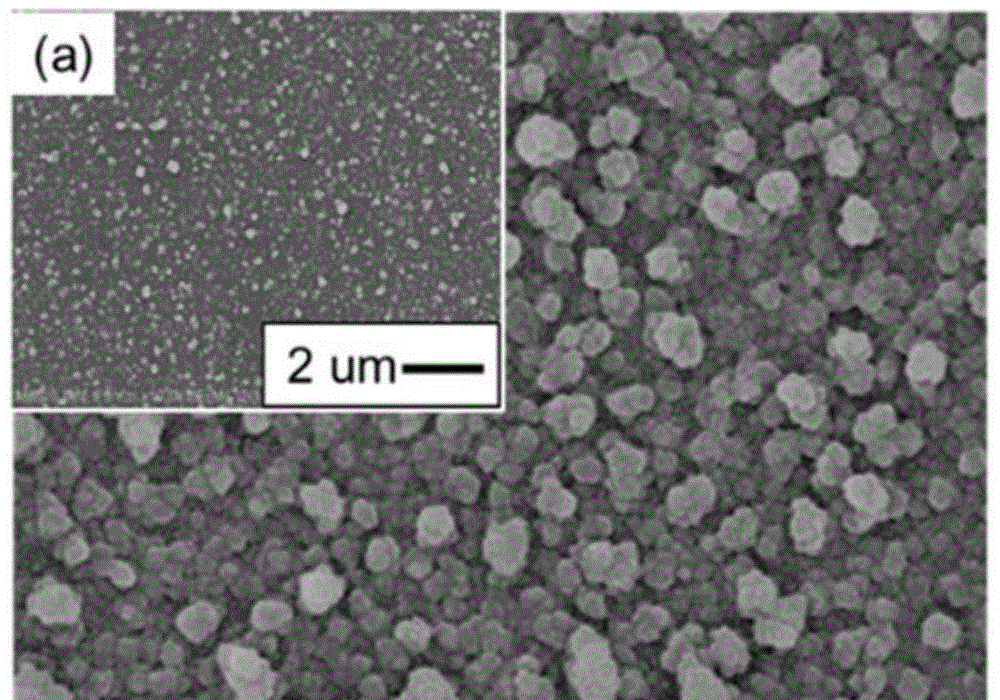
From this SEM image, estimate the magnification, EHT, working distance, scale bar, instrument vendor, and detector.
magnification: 25 K X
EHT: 5 kV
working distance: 8.4 mm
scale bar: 1000 nm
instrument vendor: Hitachi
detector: SE(M)